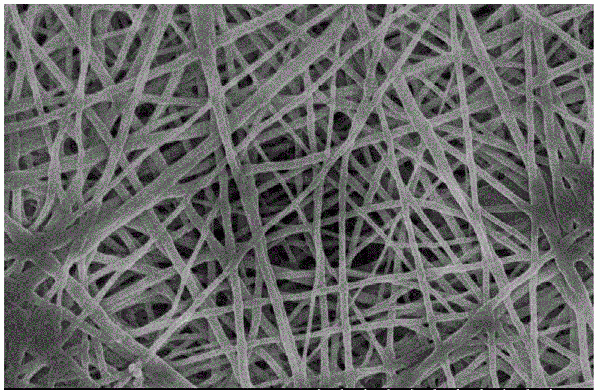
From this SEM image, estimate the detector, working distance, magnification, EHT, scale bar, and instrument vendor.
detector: SE(M)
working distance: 8.1 mm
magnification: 10 K X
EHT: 5 kV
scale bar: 5000 nm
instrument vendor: Hitachi